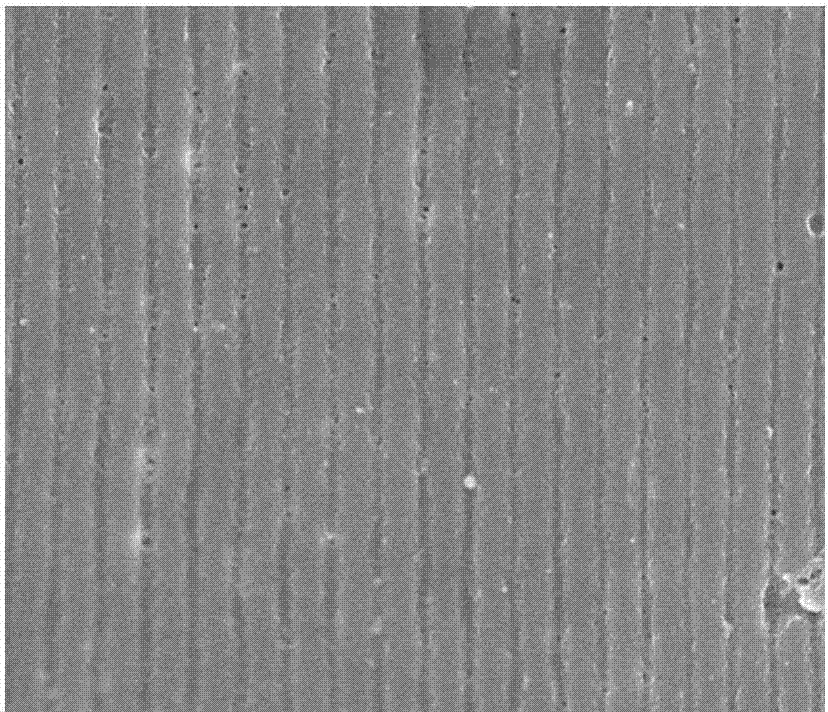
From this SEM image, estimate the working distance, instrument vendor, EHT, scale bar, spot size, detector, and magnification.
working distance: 4.3 mm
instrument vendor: FEI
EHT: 10 kV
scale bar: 5000 nm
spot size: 3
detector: TLD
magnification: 20 K X